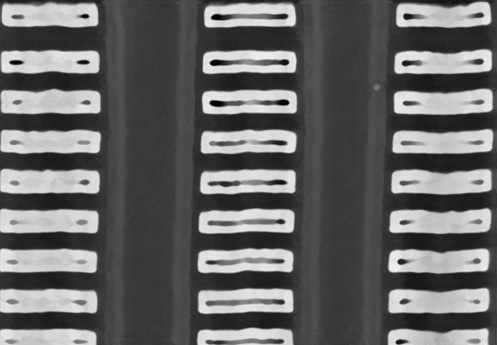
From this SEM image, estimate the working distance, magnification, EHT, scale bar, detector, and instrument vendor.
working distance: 1.5 mm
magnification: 200 K X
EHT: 1.5 kV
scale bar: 200 nm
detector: UD(F-150)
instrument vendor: Hitachi